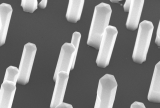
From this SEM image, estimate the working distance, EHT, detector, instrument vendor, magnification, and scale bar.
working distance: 9.9 mm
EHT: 15 kV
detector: SE(U)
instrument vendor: Hitachi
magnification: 30 K X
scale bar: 1000 nm